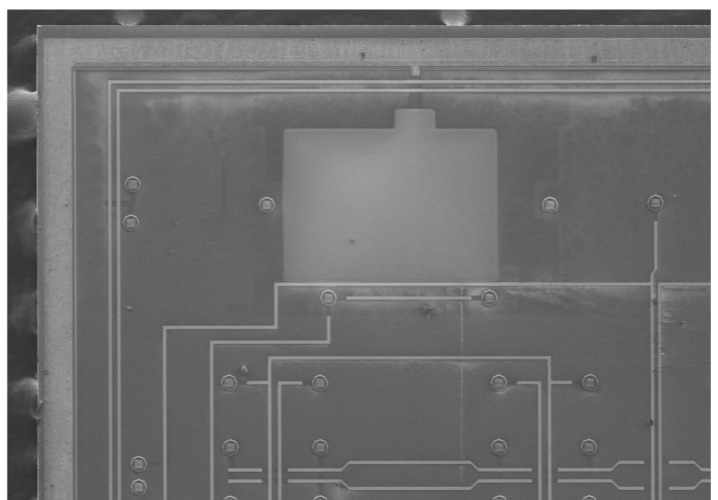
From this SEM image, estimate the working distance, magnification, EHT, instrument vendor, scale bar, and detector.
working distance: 8 mm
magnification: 0.045 K X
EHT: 3 kV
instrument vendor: Hitachi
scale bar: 1000000 nm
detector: SE(M)